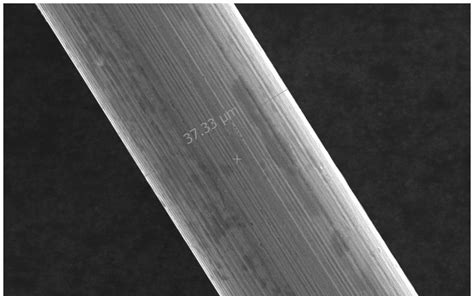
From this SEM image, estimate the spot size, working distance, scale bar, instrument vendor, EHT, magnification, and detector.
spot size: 2.5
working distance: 10.1 mm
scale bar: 20000 nm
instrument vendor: FEI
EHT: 20 kV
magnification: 2 K X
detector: ETD